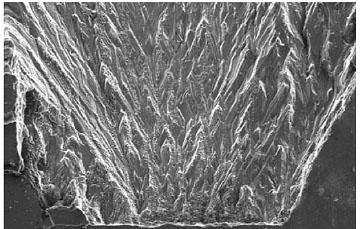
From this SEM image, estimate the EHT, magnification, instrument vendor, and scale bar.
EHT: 20 kV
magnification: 0.1 K X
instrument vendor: JEOL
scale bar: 100000 nm